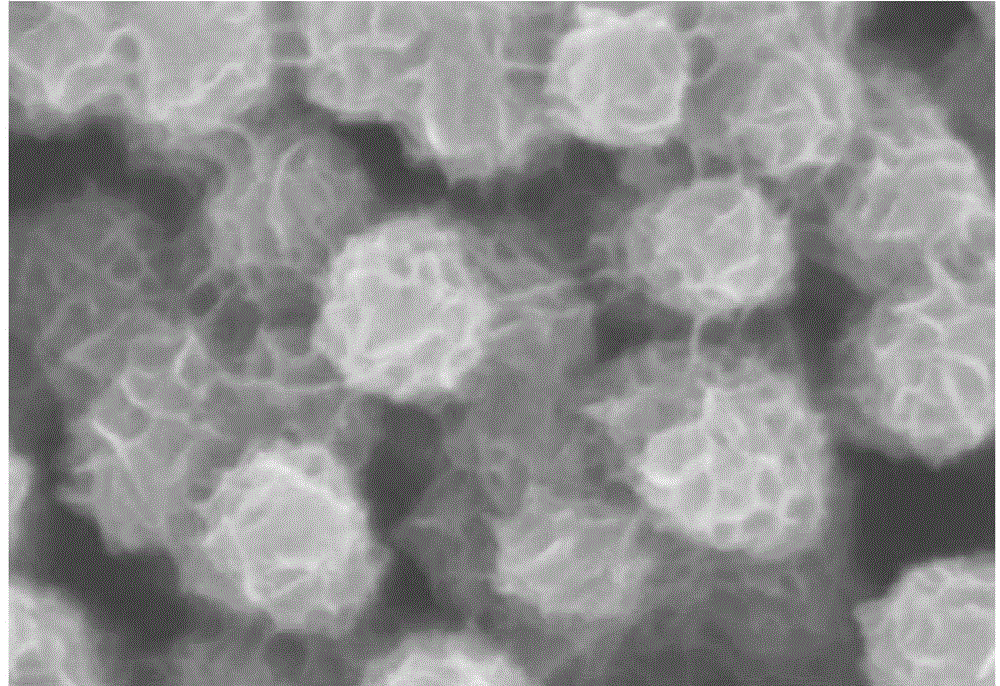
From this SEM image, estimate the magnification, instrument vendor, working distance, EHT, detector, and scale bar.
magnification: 130 K X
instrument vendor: Hitachi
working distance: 8.4 mm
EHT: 3 kV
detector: SE(M)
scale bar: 400 nm